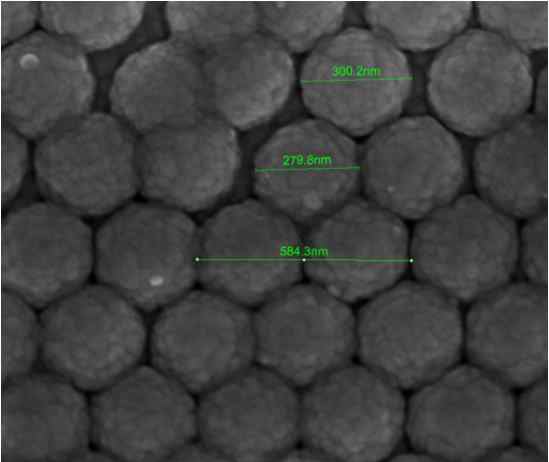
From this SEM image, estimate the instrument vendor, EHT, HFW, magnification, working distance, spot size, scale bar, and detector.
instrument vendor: FEI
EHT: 10 kV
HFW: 1.49 µm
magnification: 200 K X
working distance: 4.6 mm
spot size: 3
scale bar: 500 nm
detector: TLD